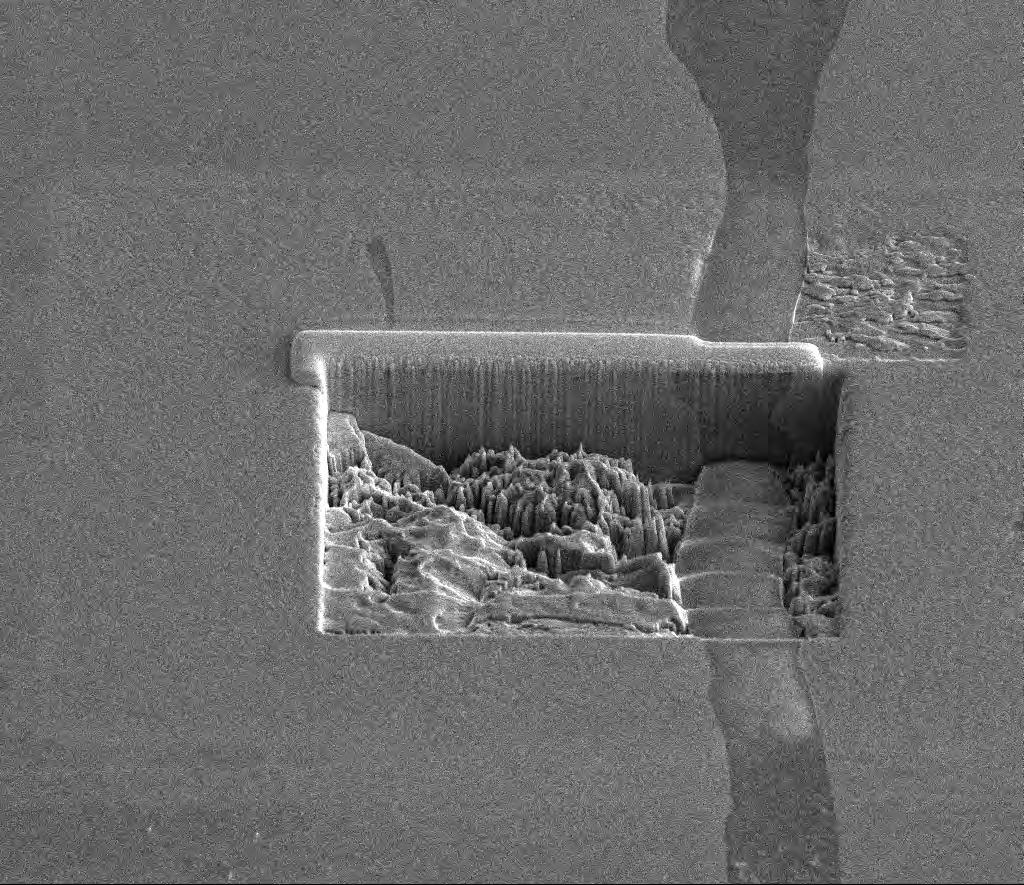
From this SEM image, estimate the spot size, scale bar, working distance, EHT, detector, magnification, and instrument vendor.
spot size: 4.5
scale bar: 20000 nm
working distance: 10 mm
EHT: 10 kV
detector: ETD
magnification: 2.5 K X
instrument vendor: FEI